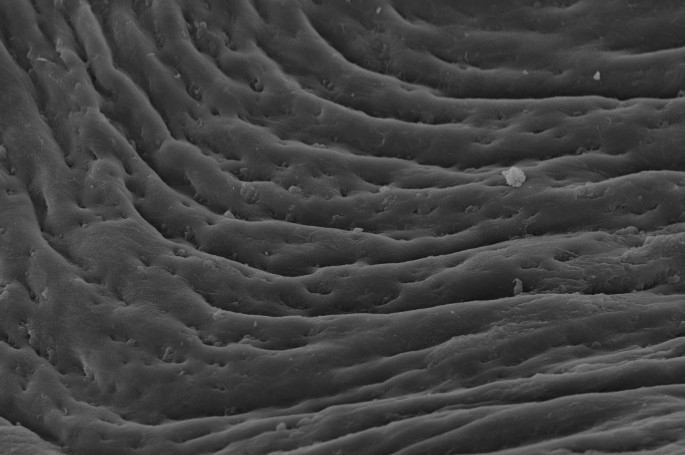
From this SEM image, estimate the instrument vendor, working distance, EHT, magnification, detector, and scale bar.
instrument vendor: Zeiss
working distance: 24 mm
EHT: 20 kV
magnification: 1 K X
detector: SE1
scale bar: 10000 nm